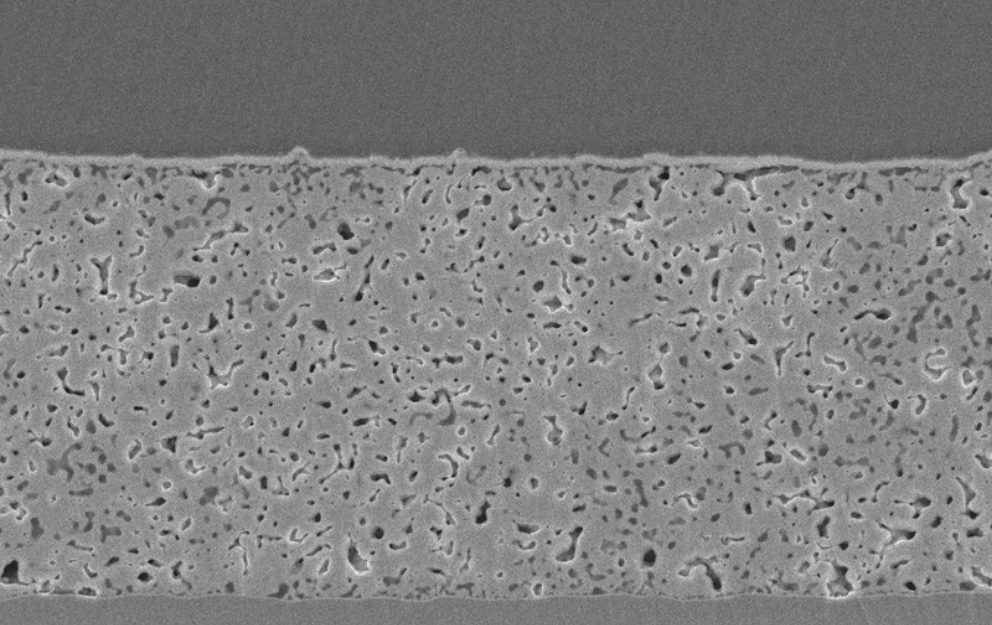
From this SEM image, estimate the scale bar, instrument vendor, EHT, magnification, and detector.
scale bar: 10000 nm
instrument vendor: JEOL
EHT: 15 kV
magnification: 2 K X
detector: SEI